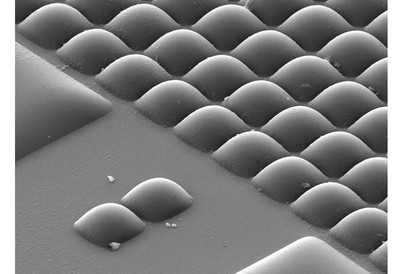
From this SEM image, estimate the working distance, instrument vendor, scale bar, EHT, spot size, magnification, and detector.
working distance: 6.4 mm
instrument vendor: FEI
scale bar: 5000 nm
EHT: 10 kV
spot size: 3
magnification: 3.5 K X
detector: SE